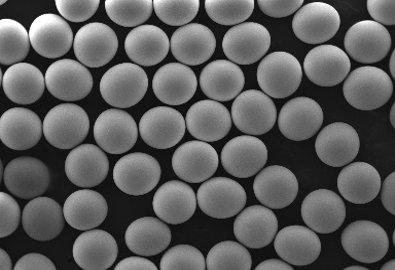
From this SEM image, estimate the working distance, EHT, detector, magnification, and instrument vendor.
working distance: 12.22 mm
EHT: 10 kV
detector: SE, BSE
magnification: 0.1 K X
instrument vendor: Tescan